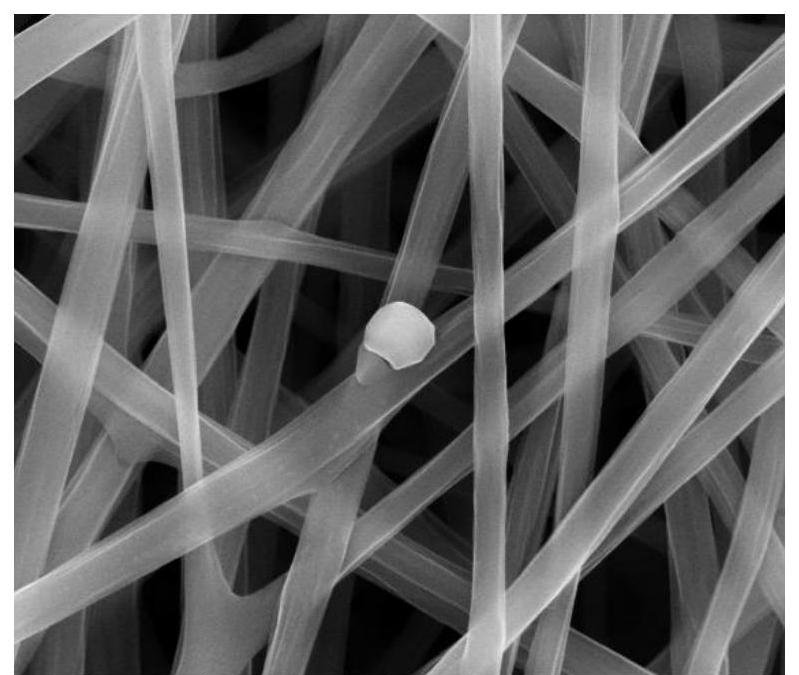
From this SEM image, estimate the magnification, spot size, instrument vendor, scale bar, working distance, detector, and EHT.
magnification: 10 K X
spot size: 3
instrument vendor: FEI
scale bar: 5000 nm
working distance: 11.1 mm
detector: ETD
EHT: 20 kV